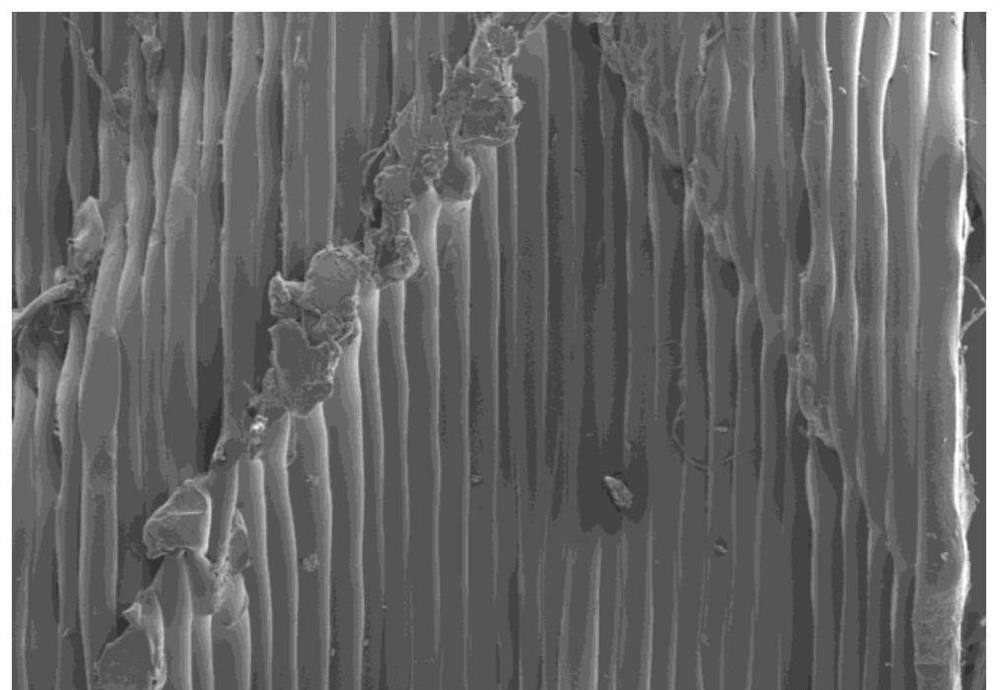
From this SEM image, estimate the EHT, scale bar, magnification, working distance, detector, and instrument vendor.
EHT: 10 kV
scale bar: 1000000 nm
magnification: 0.035 K X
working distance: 5 mm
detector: SE(M)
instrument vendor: Hitachi